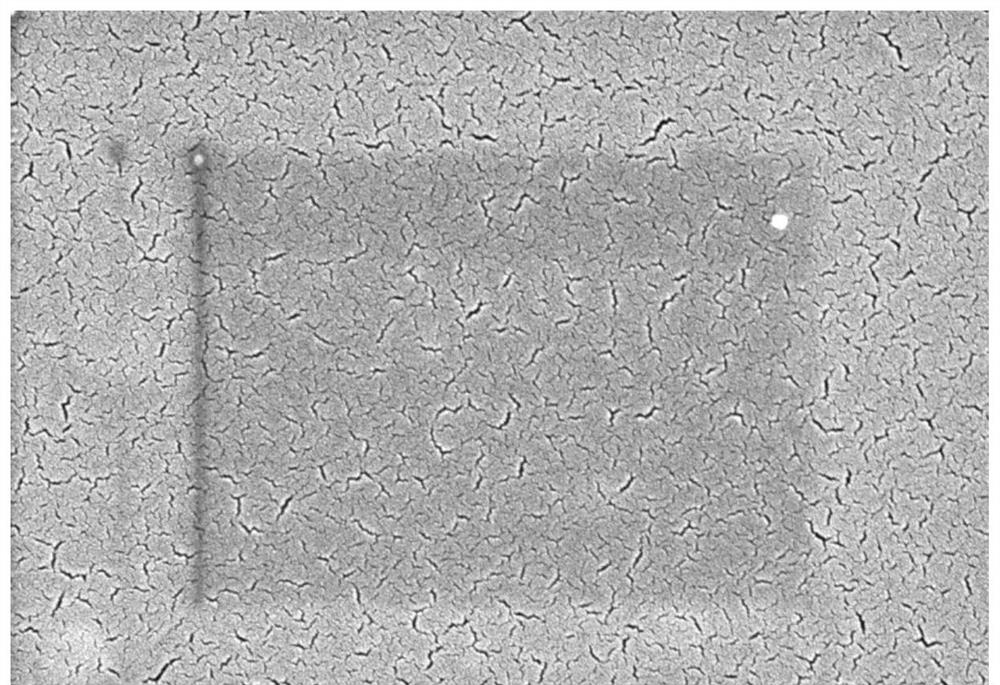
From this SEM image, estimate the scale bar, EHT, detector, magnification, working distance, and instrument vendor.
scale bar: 1000 nm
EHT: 3 kV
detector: SE(M)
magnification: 50 K X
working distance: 8 mm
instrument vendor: Hitachi